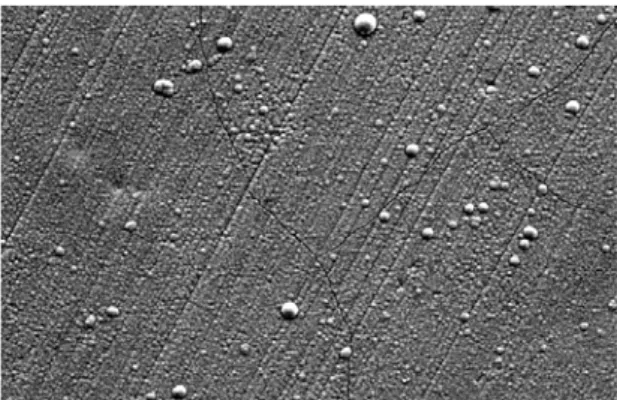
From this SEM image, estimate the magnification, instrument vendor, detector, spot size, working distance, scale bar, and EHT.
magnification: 1 K X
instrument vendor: FEI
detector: SE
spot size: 6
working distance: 10.1 mm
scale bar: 50000 nm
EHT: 20 kV